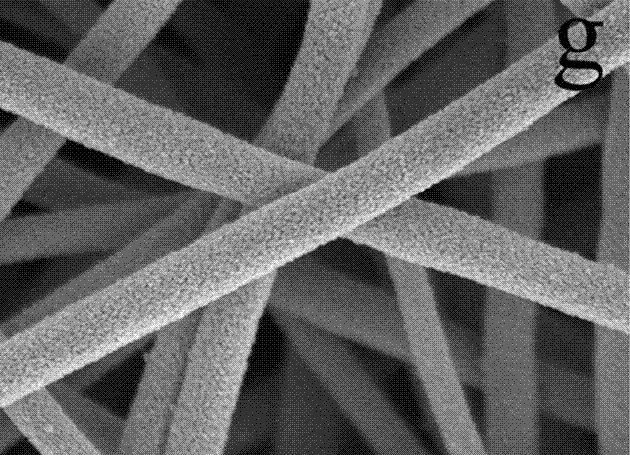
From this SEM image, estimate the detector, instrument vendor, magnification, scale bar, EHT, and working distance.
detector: InBeam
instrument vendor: Tescan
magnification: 100 K X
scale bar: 500 nm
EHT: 20 kV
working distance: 6.08 mm